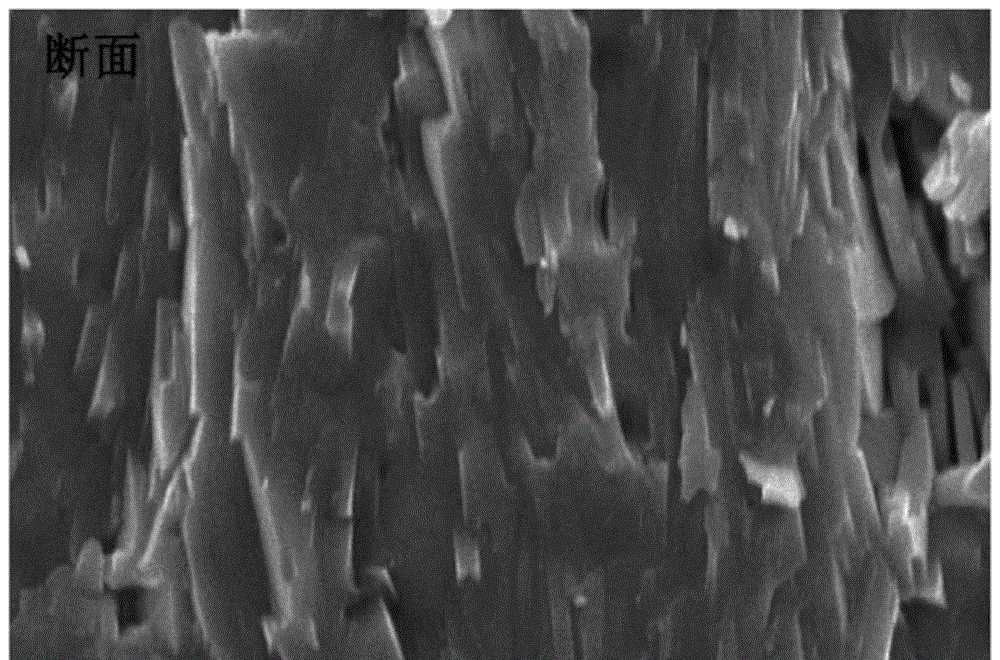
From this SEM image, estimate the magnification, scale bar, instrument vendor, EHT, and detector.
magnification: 10 K X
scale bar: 1000 nm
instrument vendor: JEOL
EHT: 20 kV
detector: SEI